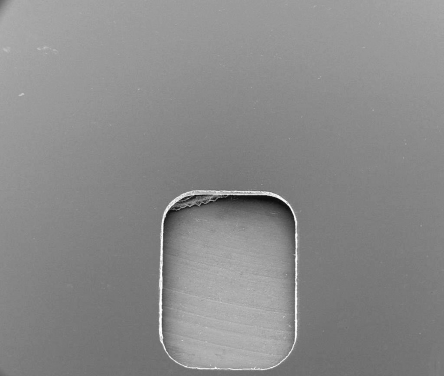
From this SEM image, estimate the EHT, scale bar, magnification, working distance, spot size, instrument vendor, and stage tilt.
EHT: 30 kV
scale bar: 2000000 nm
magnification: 0.04 K X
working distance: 18.9 mm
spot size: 5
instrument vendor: FEI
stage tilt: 25°